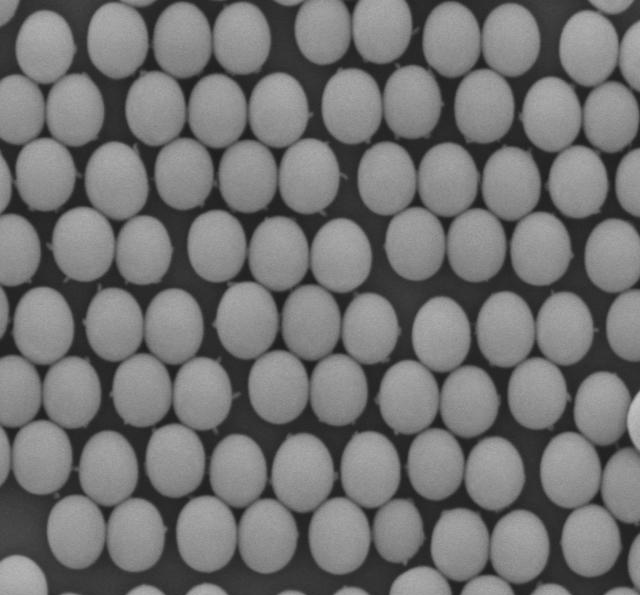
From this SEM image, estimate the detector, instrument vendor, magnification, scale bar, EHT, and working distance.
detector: LED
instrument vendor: JEOL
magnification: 30 K X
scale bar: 100 nm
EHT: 5 kV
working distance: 8 mm